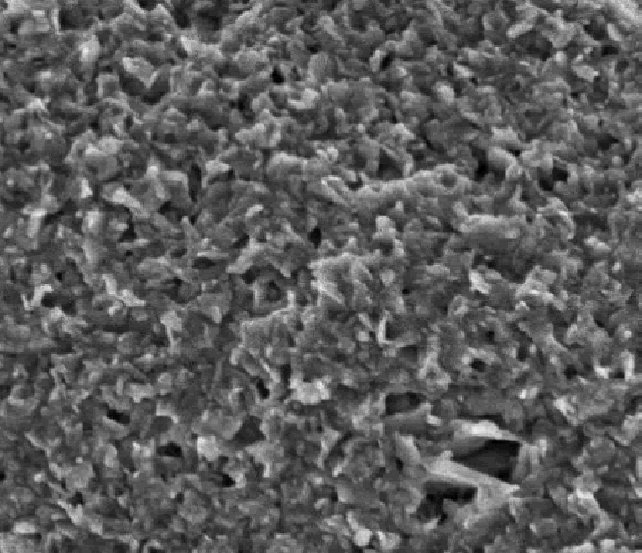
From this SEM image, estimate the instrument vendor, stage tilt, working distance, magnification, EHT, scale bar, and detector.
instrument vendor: FEI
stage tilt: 0°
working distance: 4 mm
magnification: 200 K X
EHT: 2 kV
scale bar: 500 nm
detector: TLD